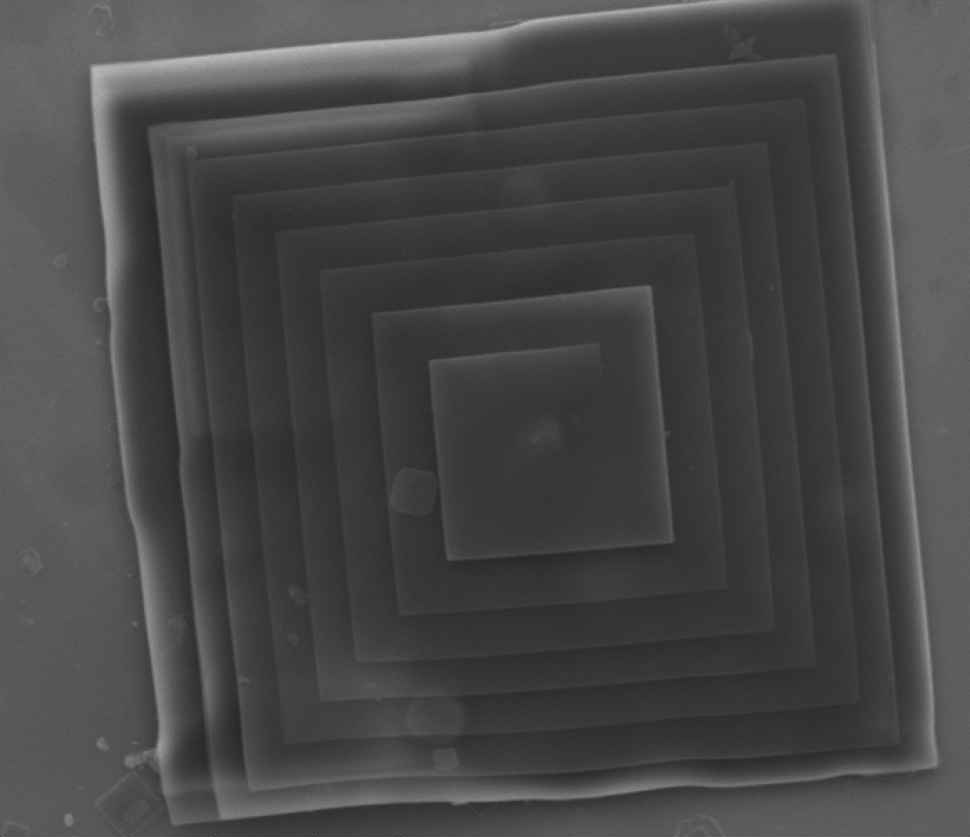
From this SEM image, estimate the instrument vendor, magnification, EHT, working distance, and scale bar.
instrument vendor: FEI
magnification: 24 K X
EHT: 5 kV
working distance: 7.4 mm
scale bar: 5000 nm